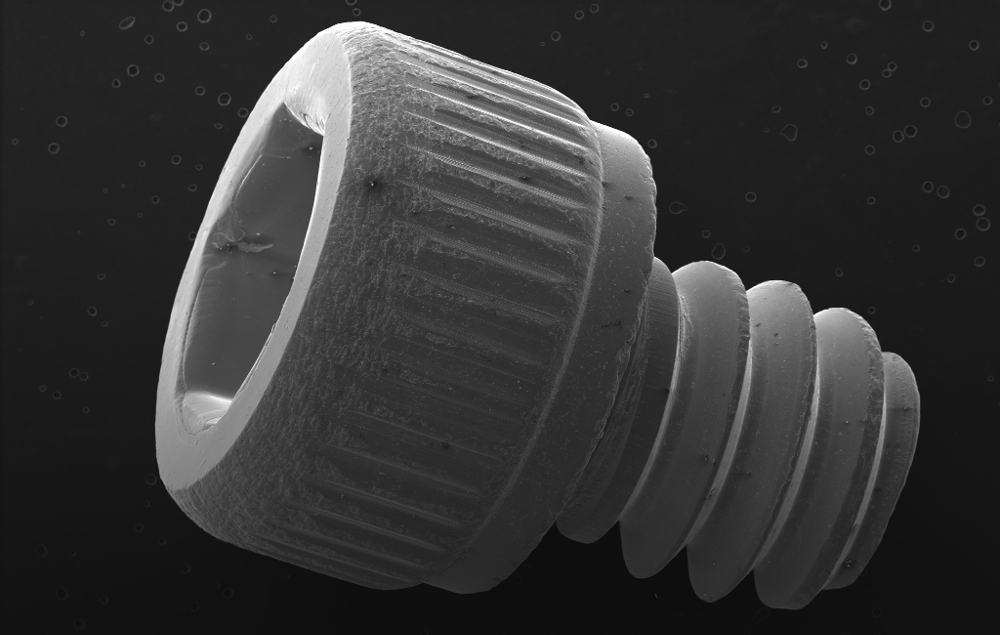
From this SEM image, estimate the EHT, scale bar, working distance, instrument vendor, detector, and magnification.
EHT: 20 kV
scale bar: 1000000 nm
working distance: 41.7 mm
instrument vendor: Zeiss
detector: SE2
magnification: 0.015 K X